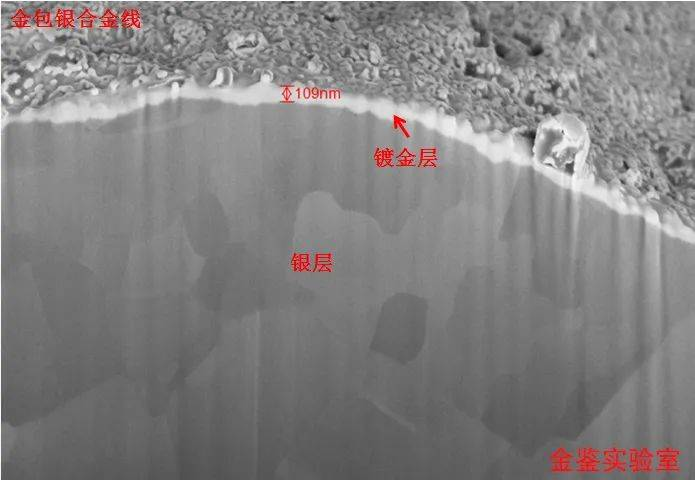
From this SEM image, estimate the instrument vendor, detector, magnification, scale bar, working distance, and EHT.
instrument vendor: Zeiss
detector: InLens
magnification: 25 K X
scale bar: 200 nm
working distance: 4.1 mm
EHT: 5 kV